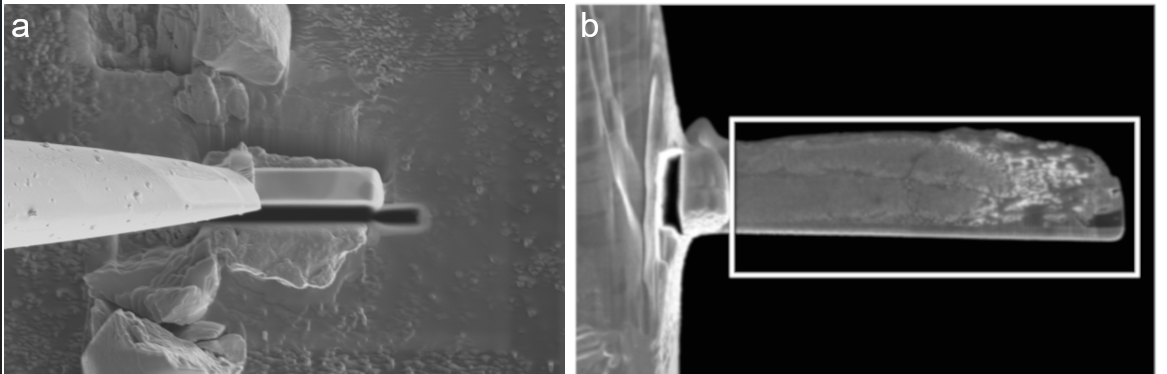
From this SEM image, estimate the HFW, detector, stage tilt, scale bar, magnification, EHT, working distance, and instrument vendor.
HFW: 26 µm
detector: ETD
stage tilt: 0°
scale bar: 10000 nm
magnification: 4.878 K X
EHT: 2 kV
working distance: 3.9 mm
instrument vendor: Thermo